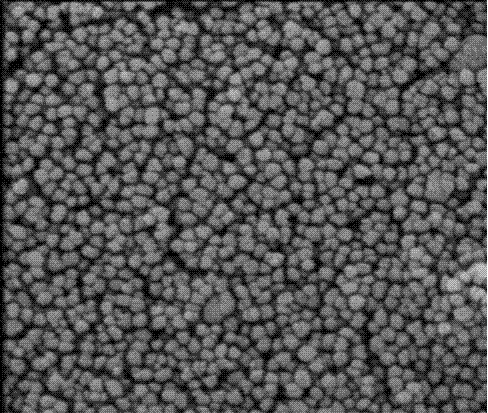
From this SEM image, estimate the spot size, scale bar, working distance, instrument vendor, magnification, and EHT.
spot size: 3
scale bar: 500 nm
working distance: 4.5 mm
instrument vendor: FEI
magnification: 80 K X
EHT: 3 kV